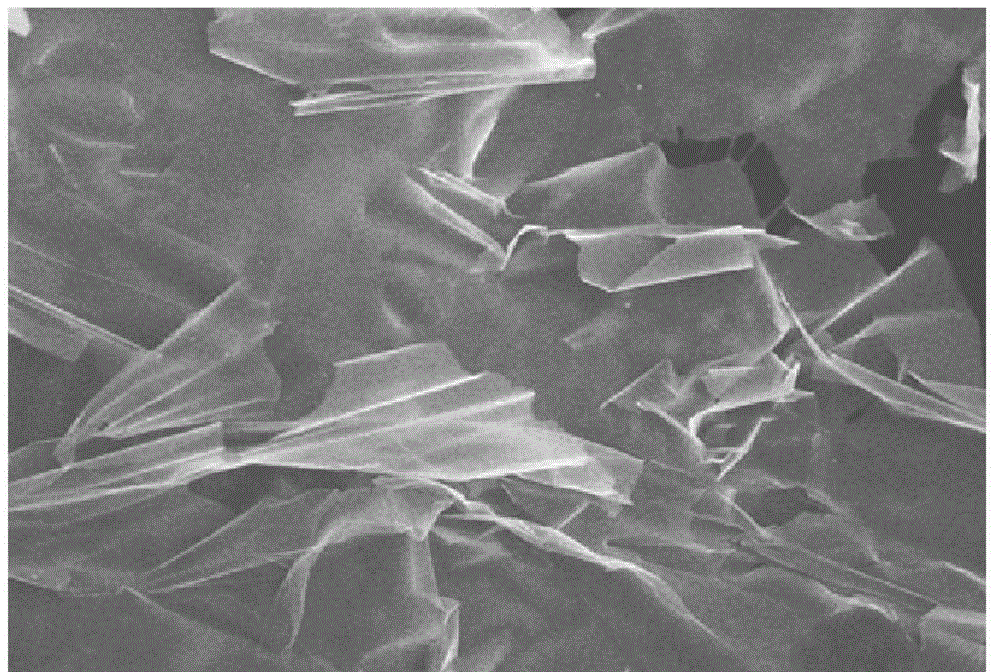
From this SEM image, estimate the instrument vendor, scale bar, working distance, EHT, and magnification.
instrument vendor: Hitachi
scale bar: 20000 nm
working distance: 9.6 mm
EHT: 10 kV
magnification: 2 K X